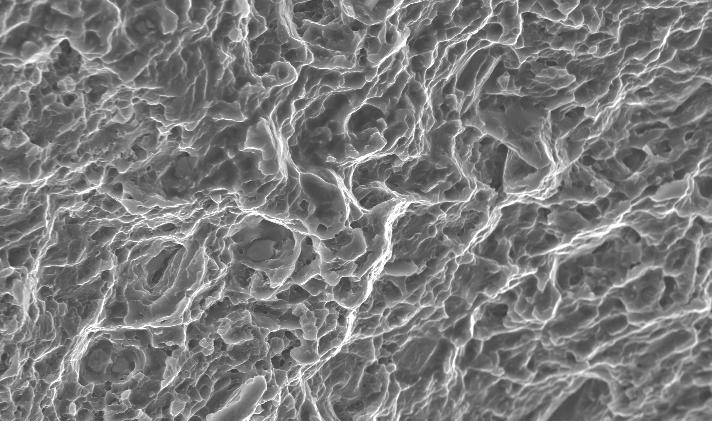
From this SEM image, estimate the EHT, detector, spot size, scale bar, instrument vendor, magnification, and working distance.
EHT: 20 kV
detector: SE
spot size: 4.1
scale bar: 50000 nm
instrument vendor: FEI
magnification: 0.4 K X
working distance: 16.8 mm